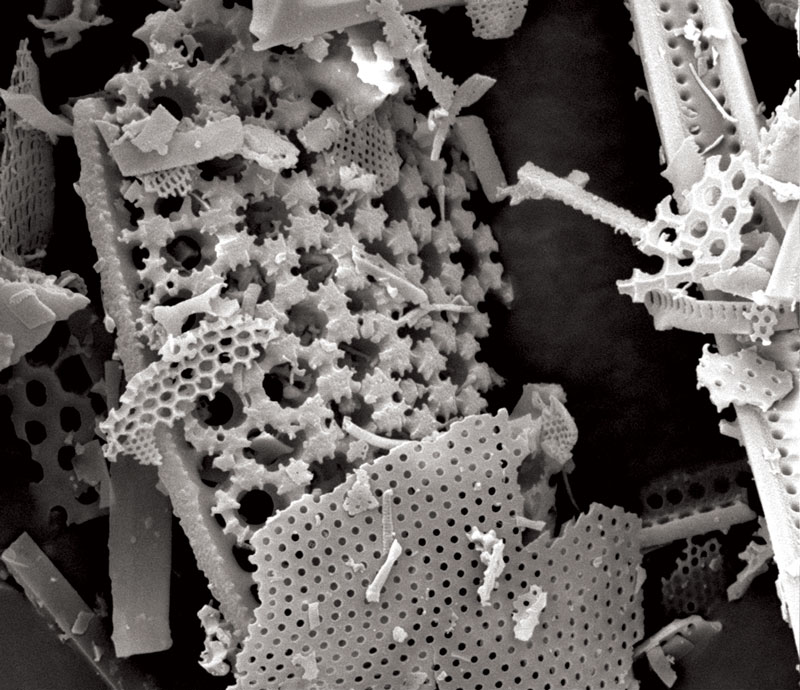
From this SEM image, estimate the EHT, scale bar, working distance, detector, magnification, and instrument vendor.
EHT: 10 kV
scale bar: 10000 nm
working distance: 9.6 mm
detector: ETD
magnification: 10 K X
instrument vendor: FEI